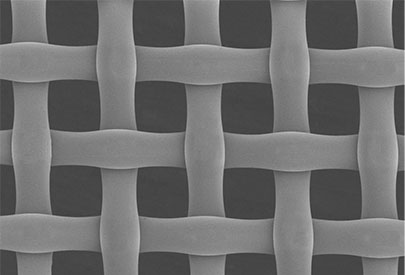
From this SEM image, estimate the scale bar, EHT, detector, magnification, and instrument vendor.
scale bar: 100000 nm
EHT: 1 kV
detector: SE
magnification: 0.35 K X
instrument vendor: Hitachi